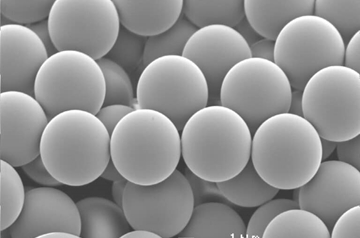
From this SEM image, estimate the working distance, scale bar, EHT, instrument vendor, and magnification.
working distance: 18 mm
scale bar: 1000 nm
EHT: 5 kV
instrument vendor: JEOL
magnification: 5 K X